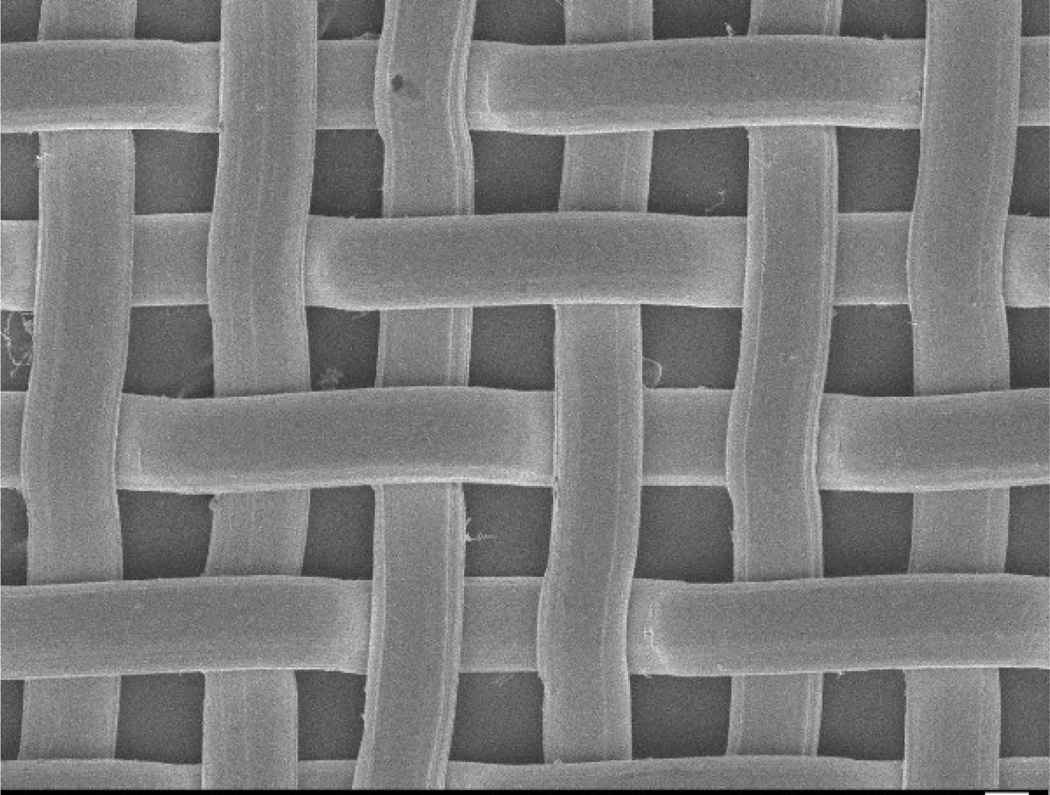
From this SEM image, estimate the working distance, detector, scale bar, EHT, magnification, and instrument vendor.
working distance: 7.5 mm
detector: SEI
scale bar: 10000 nm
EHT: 5 kV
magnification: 0.5 K X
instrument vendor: JEOL